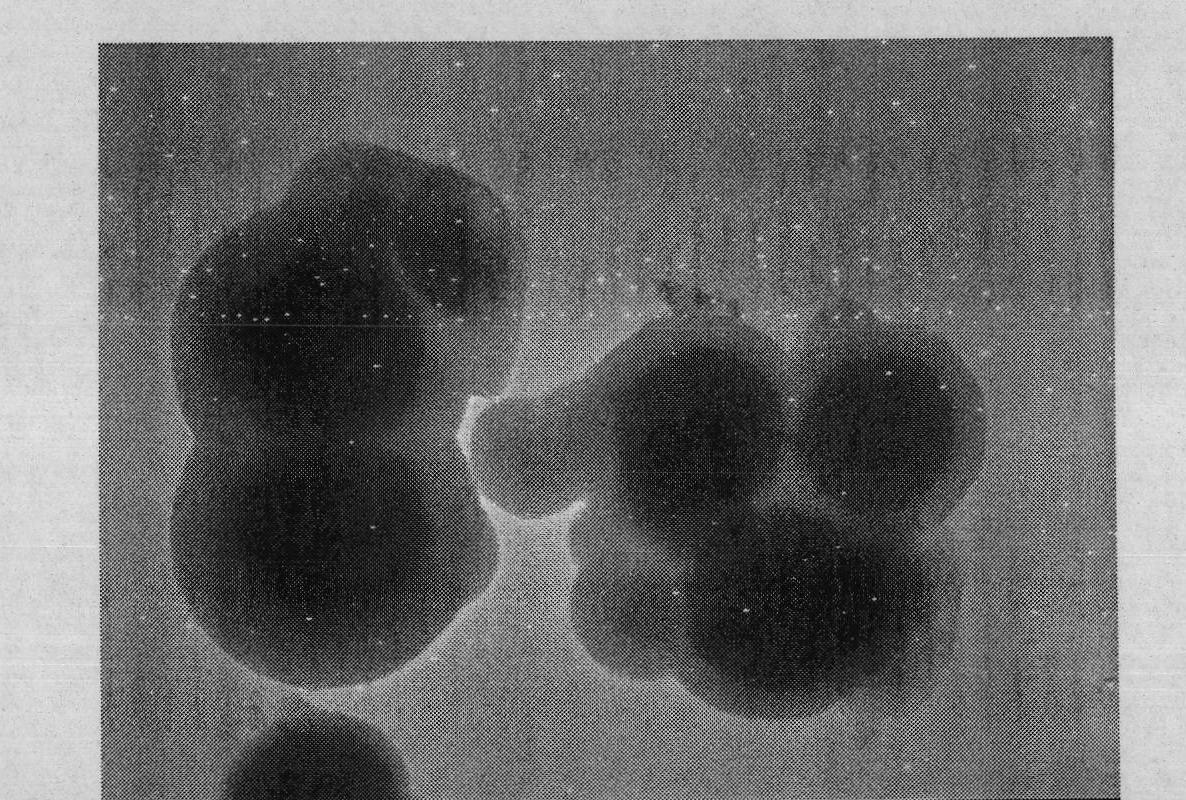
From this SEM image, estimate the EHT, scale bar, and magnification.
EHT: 200 kV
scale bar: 400 nm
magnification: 50 K X